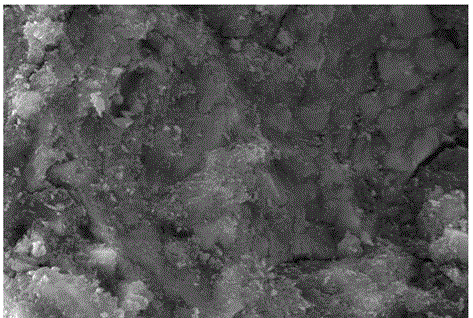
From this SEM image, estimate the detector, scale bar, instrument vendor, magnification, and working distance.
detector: SE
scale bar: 10000 nm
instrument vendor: Tescan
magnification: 3 K X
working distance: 7.94 mm